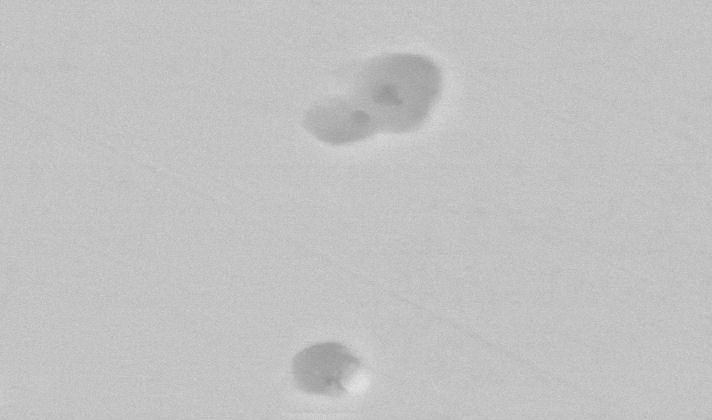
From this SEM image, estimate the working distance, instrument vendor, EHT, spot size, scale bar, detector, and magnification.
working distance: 10.8 mm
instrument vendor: FEI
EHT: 20 kV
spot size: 3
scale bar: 2000 nm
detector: BSE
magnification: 16 K X